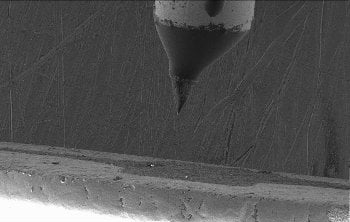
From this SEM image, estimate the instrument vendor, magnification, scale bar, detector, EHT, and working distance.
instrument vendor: Hitachi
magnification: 0.045 K X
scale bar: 1000000 nm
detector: SE(M)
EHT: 10 kV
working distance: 14.9 mm